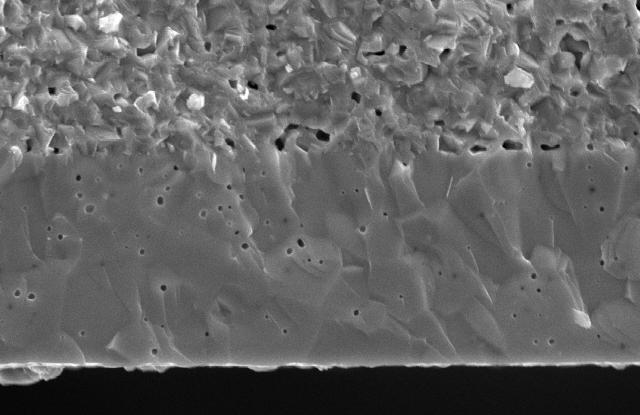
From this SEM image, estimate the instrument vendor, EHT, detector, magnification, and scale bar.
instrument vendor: JEOL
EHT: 20 kV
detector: SEI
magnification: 2.5 K X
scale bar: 10000 nm